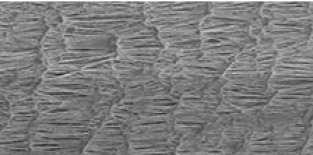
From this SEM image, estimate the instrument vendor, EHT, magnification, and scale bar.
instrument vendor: JEOL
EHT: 10 kV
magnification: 0.3 K X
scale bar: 50000 nm